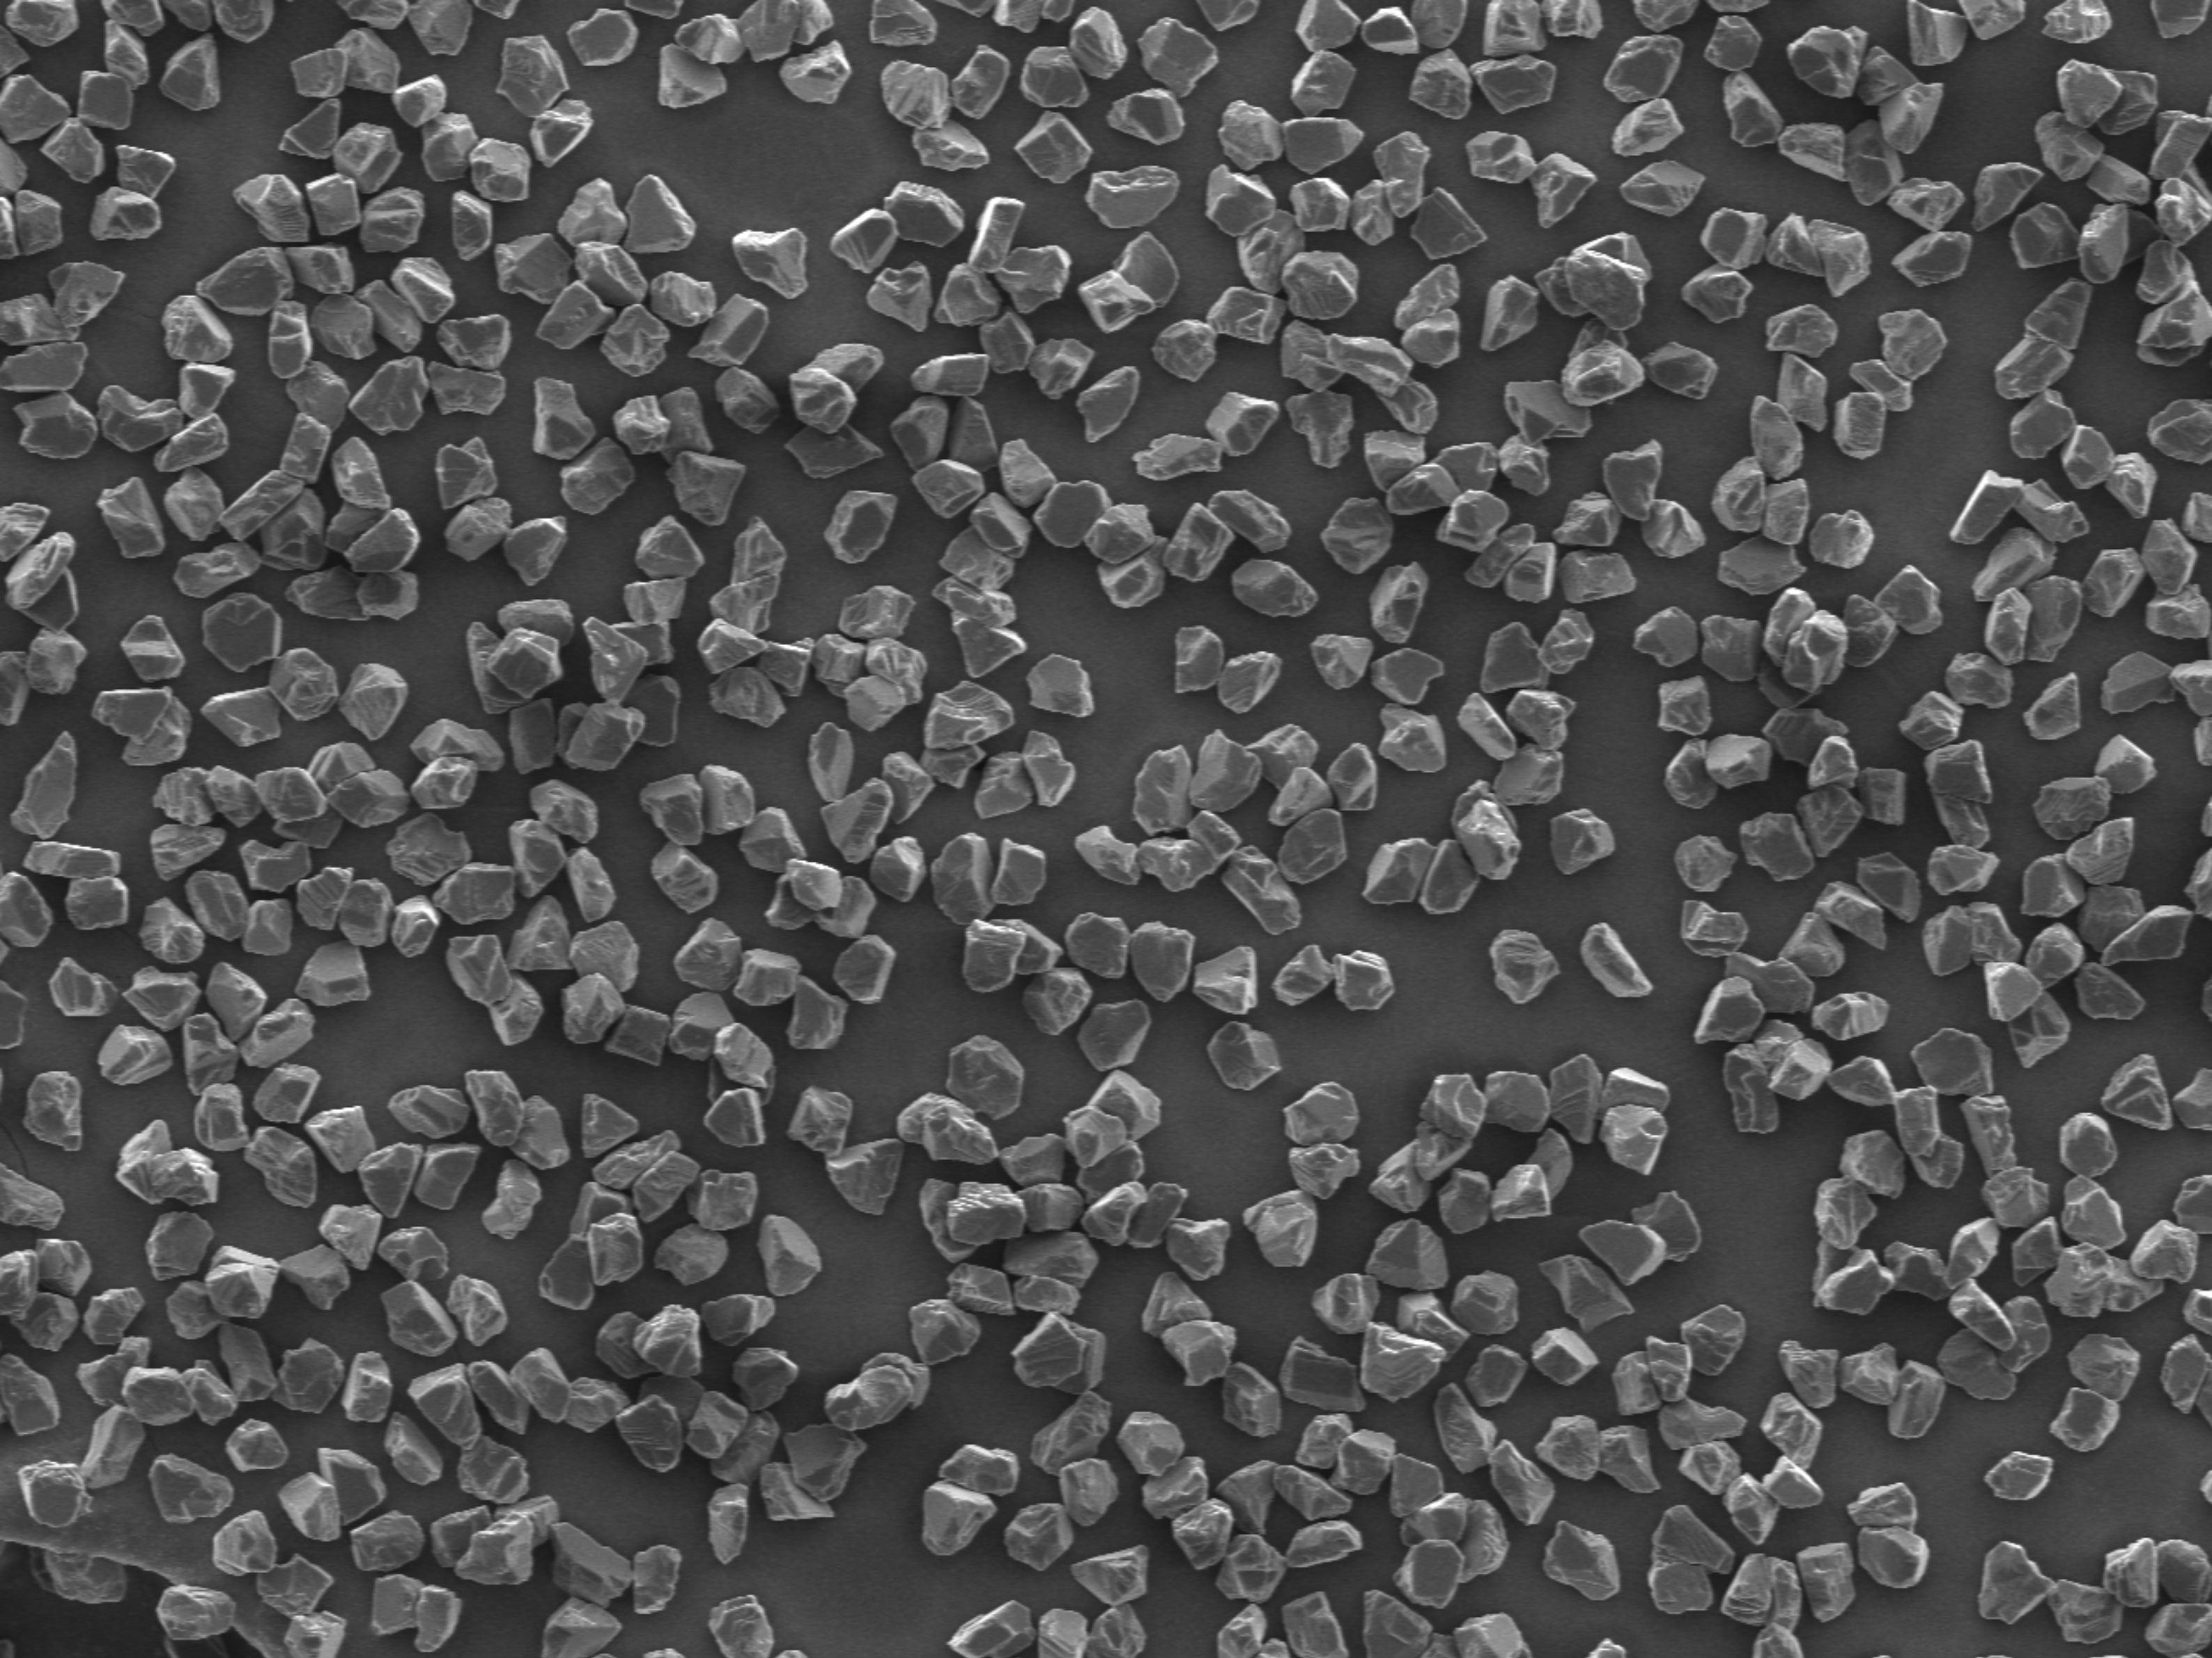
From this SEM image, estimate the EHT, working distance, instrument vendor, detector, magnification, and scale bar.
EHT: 10 kV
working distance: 9.5 mm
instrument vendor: JEOL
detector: SEI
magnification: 0.3 K X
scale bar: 10000 nm